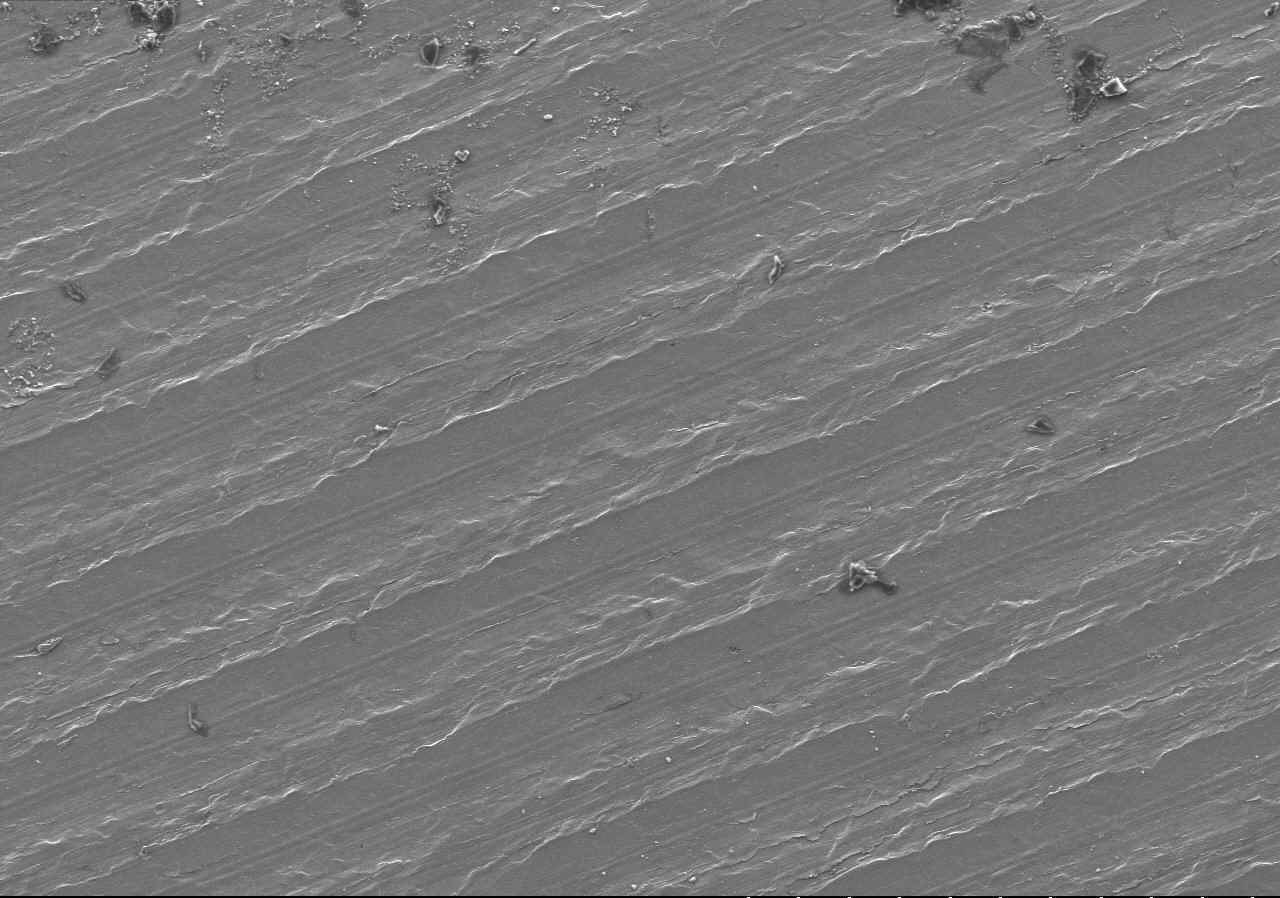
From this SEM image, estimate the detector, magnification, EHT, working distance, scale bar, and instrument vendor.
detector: SE(M)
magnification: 0.1 K X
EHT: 15 kV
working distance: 12 mm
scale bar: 500000 nm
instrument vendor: Hitachi